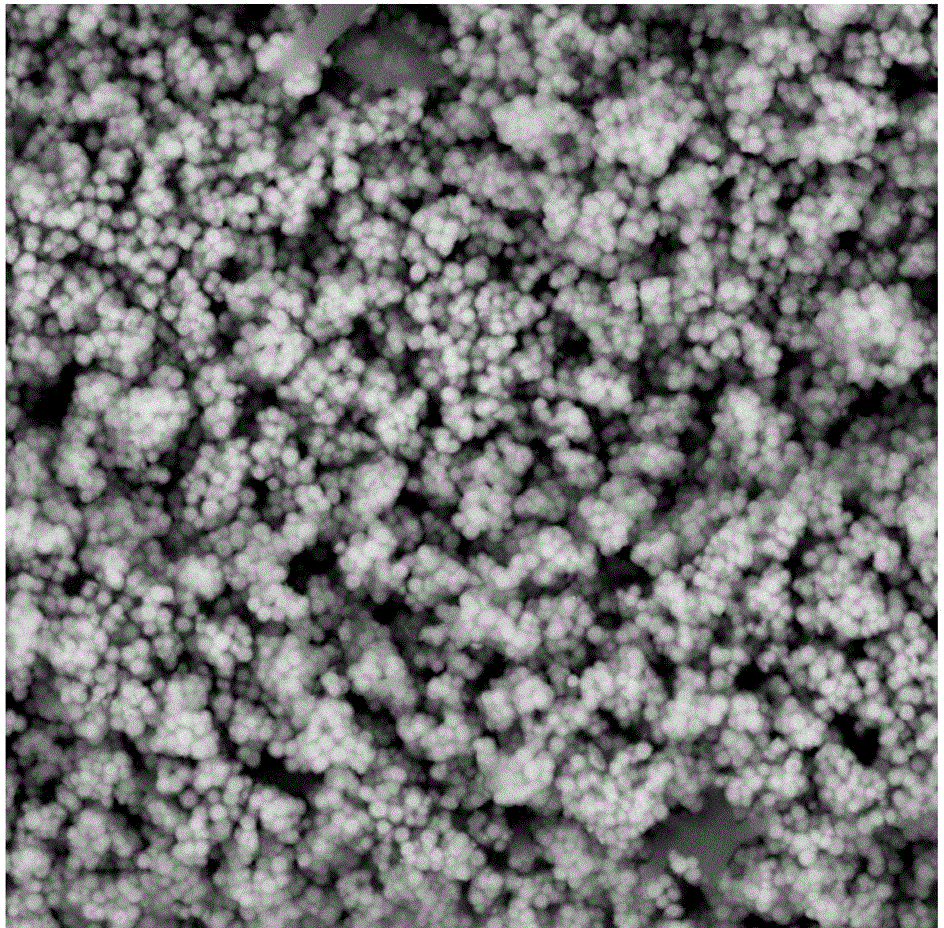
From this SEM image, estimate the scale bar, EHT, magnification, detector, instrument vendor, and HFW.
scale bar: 8000 nm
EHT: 15 kV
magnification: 10 K X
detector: SH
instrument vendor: Phenom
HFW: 26.9 µm